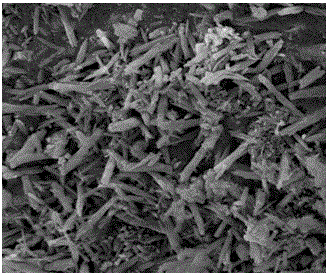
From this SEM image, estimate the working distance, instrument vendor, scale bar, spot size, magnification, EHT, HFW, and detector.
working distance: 8.2 mm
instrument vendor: FEI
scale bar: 2000 nm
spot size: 2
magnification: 20 K X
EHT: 10 kV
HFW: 7.46 µm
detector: ETD